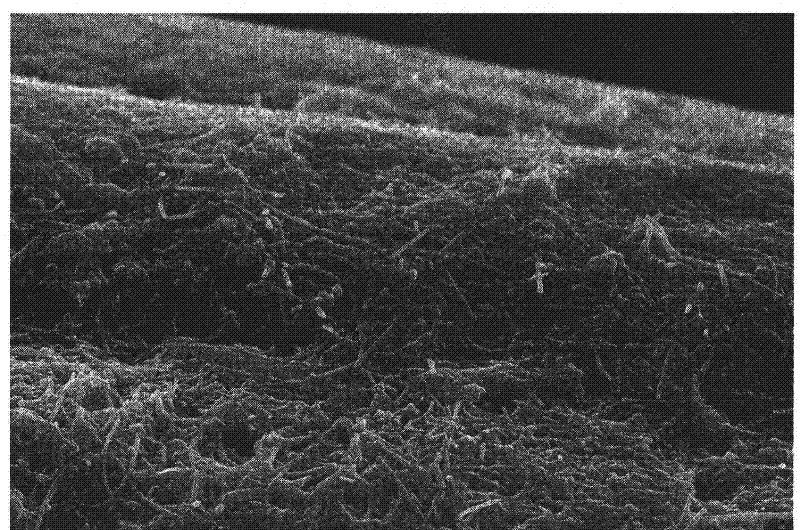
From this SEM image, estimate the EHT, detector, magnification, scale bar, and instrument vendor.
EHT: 10 kV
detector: SEI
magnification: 5 K X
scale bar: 5000 nm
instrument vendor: JEOL